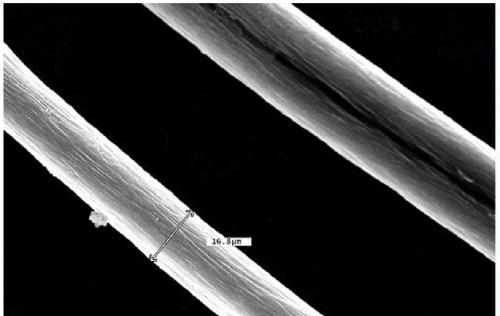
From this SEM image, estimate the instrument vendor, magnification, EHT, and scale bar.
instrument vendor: JEOL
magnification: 1 K X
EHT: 25 kV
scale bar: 10000 nm